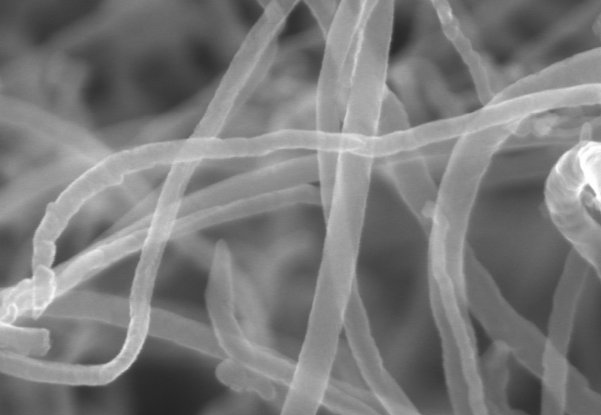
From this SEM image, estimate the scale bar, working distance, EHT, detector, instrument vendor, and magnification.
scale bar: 500 nm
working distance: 6.4 mm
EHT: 20 kV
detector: SE(M)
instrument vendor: Hitachi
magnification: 100 K X